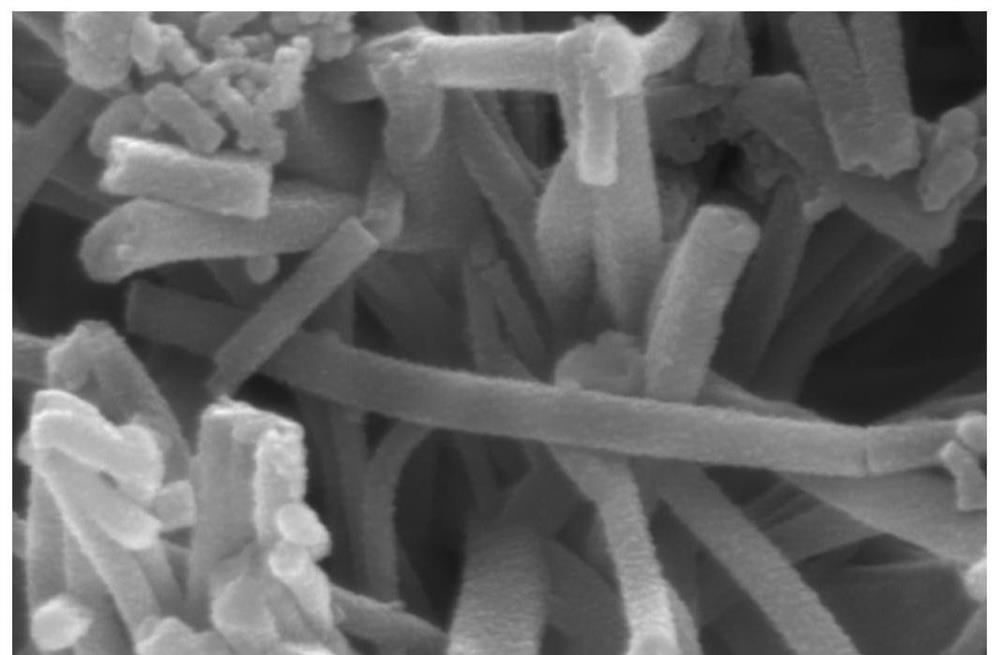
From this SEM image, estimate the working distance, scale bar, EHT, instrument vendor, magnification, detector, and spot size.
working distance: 5.3 mm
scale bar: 500 nm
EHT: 20 kV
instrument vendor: FEI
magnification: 100 K X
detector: ETD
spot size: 3